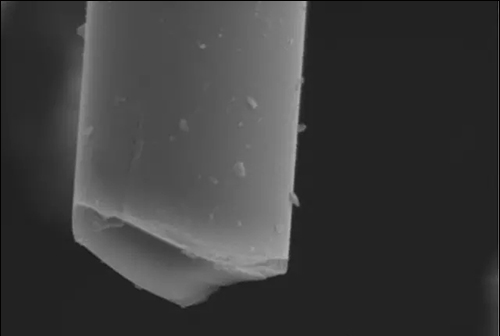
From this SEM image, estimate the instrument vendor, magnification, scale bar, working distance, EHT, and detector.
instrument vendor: Hitachi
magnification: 4 K X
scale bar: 10000 nm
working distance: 8 mm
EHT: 20 kV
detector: SE